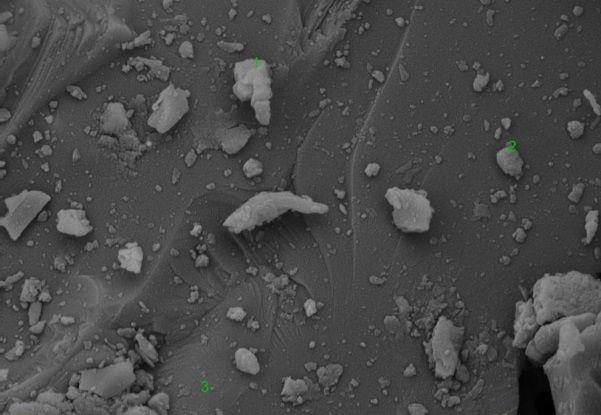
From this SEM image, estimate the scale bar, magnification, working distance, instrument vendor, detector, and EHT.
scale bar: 2000 nm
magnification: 20 K X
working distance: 7.7 mm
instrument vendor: Tescan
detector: SE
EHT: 10 kV